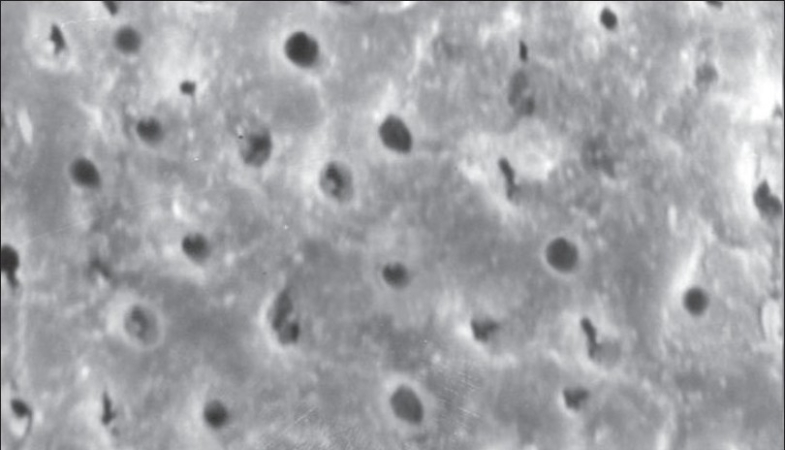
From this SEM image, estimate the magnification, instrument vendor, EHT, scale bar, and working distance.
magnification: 3.5 K X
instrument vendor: JEOL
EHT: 20 kV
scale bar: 10000 nm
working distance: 14 mm